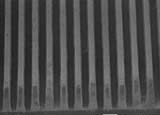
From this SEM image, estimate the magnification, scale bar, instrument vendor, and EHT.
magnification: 0.1 K X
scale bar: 100000 nm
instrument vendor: JEOL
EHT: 15 kV